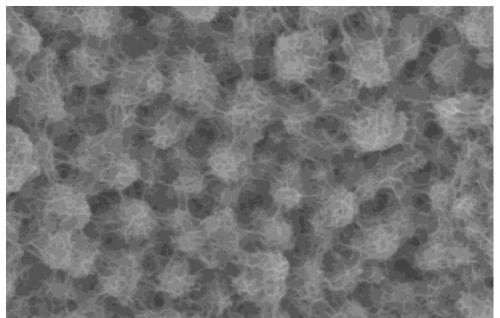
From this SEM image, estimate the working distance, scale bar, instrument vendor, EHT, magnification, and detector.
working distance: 16.7 mm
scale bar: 1000 nm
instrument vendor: Hitachi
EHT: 5 kV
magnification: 35 K X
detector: SE(U)